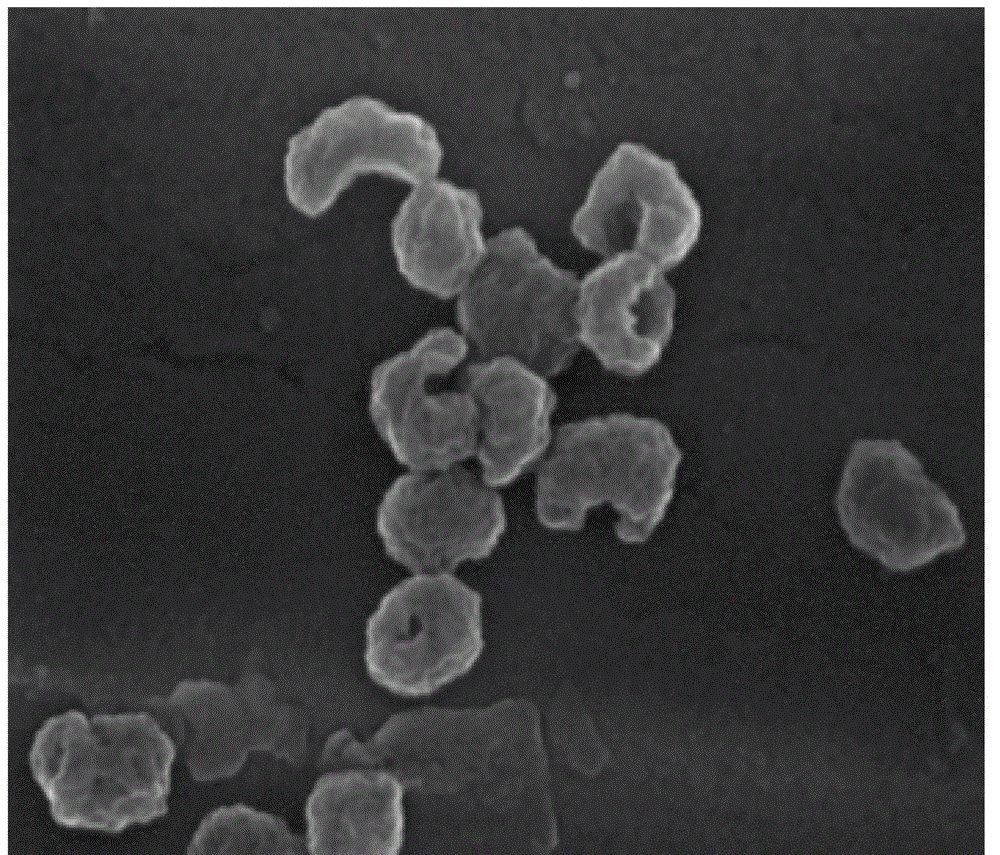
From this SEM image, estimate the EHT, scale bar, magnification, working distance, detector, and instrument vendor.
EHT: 10 kV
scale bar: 200 nm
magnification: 200 K X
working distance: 5.8 mm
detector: TLD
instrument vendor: FEI